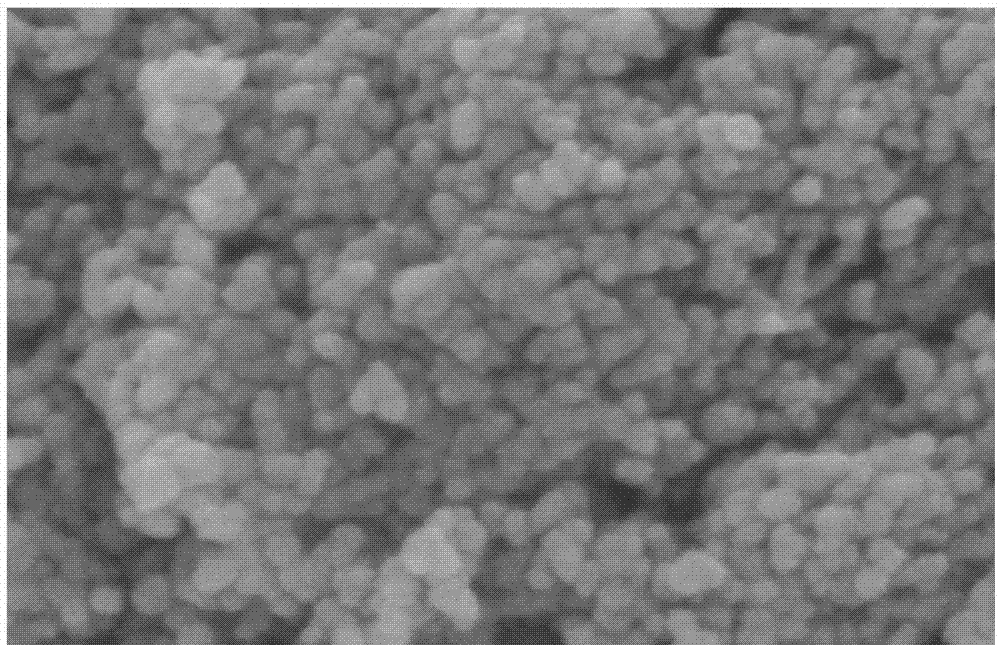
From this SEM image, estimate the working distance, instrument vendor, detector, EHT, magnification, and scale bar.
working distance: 7.9 mm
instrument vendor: Hitachi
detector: SE(U)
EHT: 5 kV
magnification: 200 K X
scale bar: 200 nm